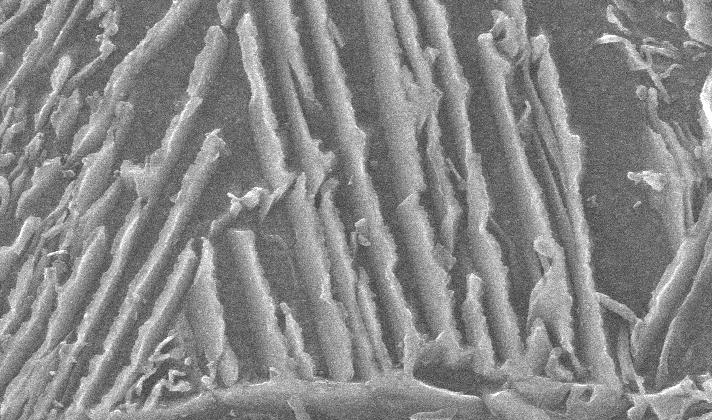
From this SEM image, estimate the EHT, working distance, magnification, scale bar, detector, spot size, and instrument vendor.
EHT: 25 kV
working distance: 8.6 mm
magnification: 20 K X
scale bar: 1000 nm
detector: SE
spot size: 3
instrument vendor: FEI